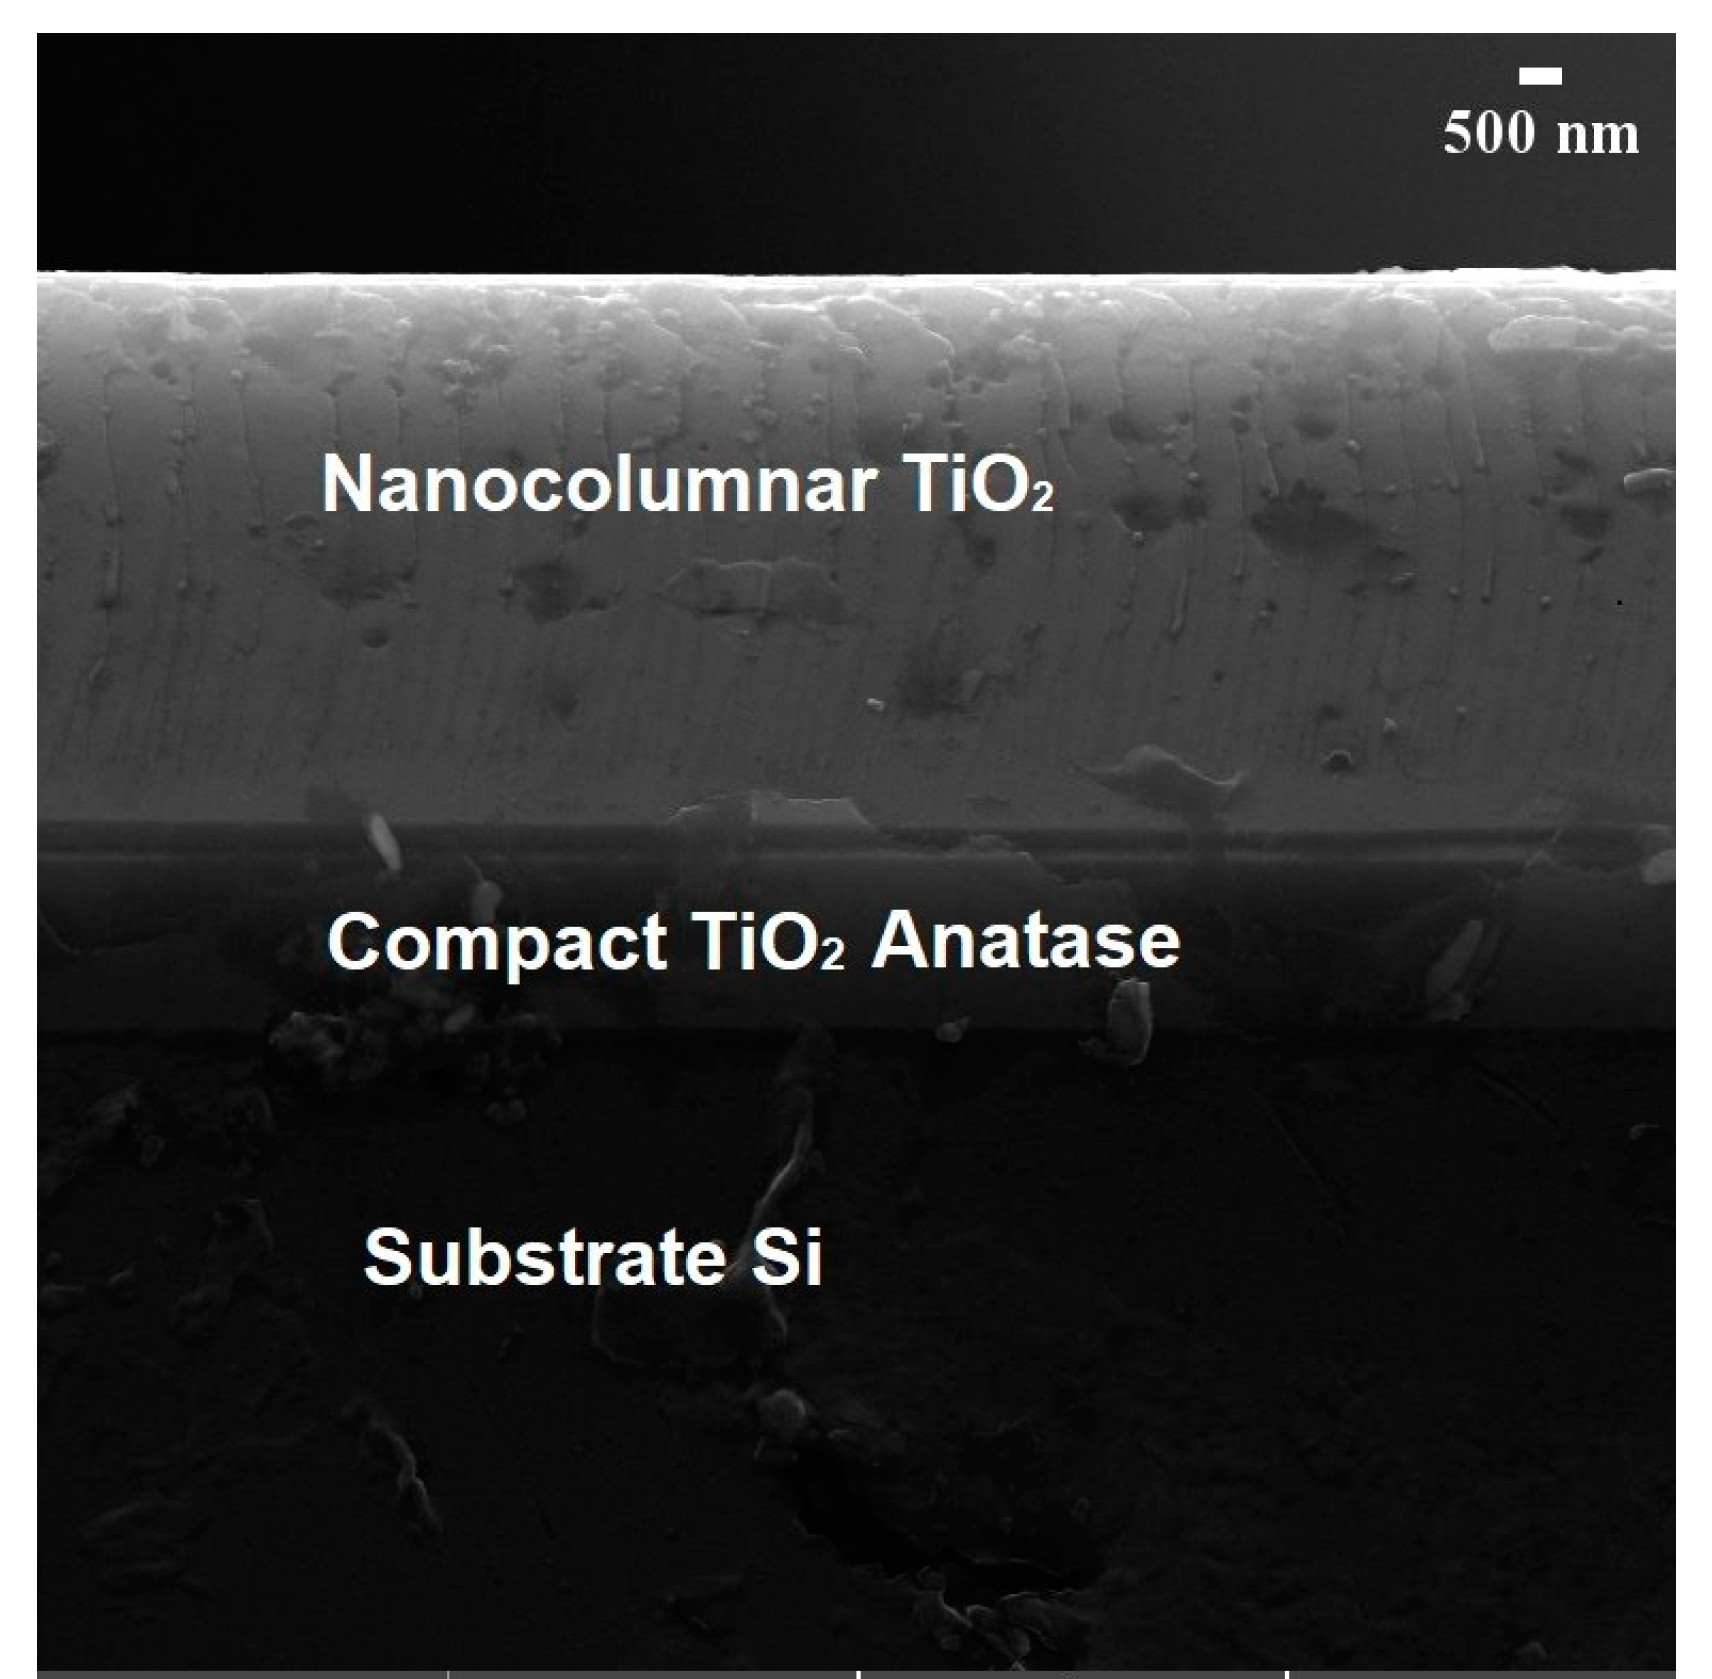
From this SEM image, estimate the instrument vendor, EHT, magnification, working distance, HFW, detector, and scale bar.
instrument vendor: Tescan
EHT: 20 kV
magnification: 10 K X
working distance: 11.65 mm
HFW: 19.1 µm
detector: SE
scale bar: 5000 nm